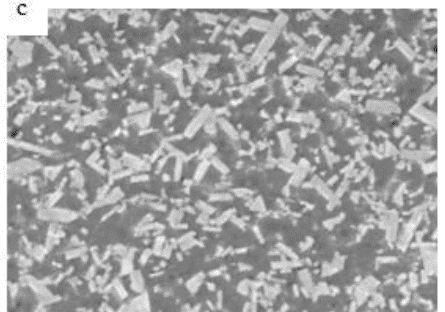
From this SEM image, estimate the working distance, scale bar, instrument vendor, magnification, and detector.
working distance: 9.6 mm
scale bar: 10000 nm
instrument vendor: Hitachi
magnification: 4 K X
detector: SE